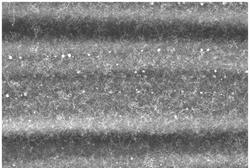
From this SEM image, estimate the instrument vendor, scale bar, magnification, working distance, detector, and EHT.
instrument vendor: Hitachi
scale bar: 10000 nm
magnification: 5 K X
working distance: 6.1 mm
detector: SE(L)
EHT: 10 kV